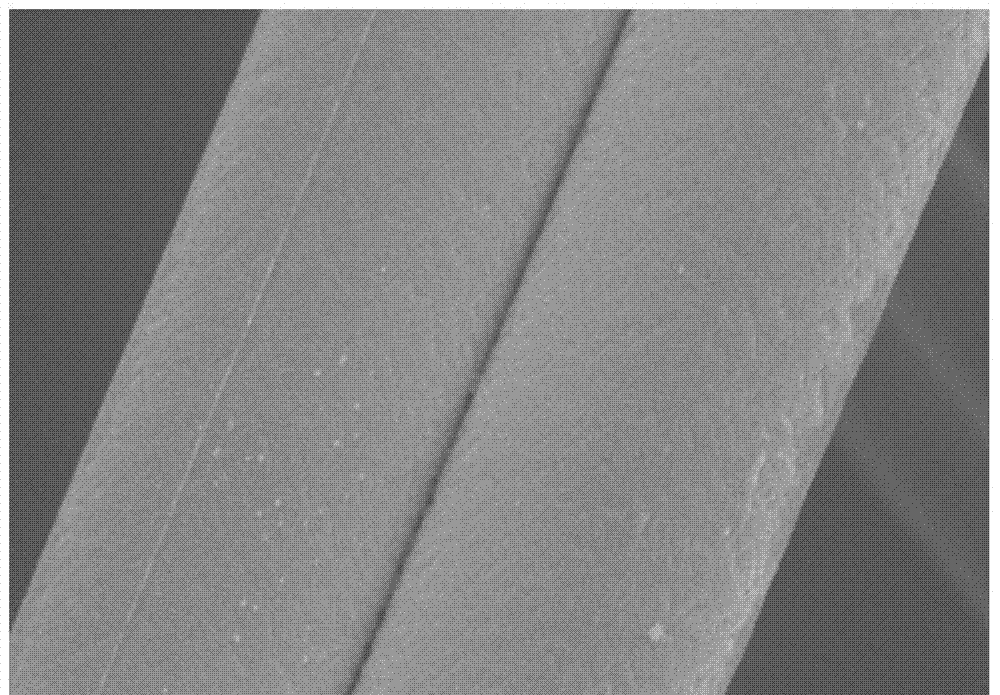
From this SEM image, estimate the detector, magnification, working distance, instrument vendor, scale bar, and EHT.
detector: SE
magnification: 4 K X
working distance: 10.6 mm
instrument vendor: Hitachi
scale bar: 10000 nm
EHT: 5 kV